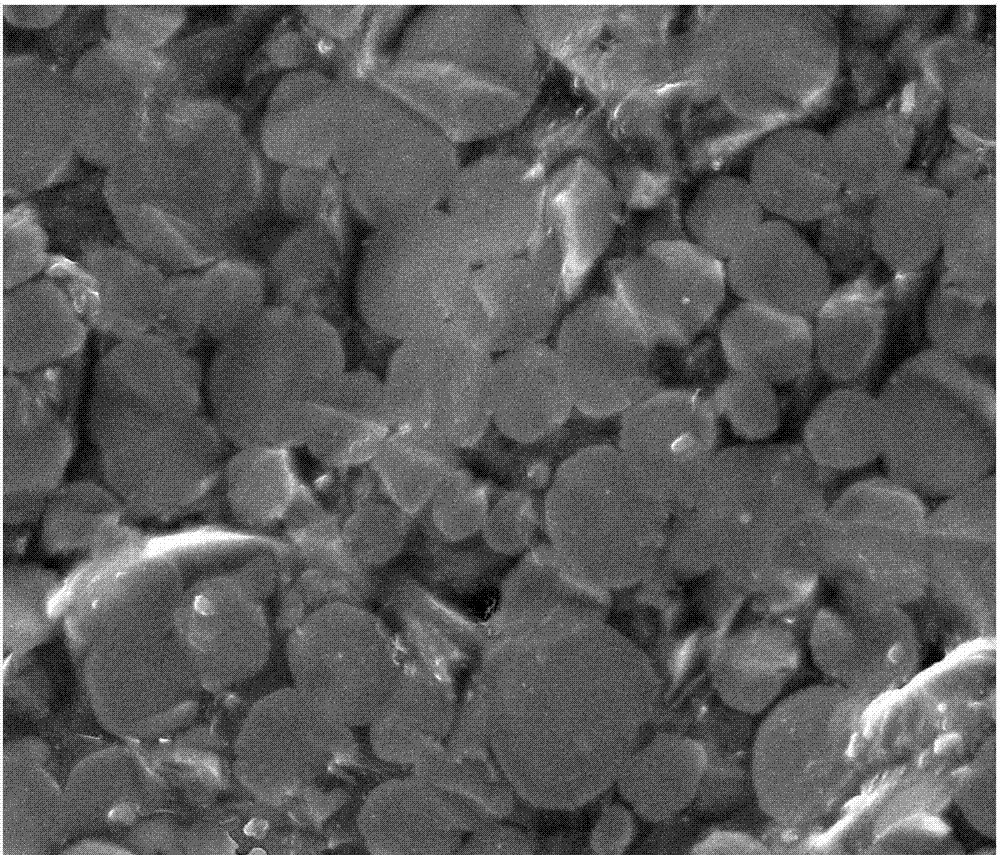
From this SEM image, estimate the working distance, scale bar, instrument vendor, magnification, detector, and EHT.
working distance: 7.5 mm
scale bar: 10000 nm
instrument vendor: FEI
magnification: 6 K X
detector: SE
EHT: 20 kV